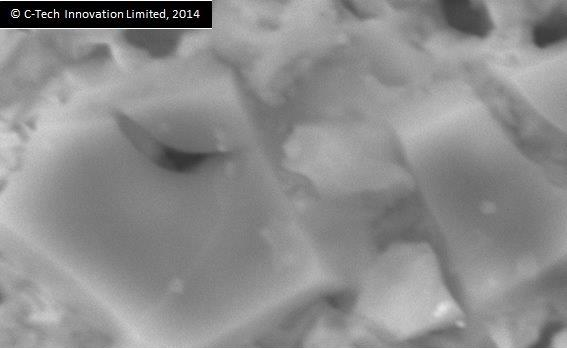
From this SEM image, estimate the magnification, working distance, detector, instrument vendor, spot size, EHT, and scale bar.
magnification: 10 K X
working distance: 10 mm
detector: SEI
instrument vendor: JEOL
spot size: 50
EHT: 20 kV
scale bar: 1000 nm